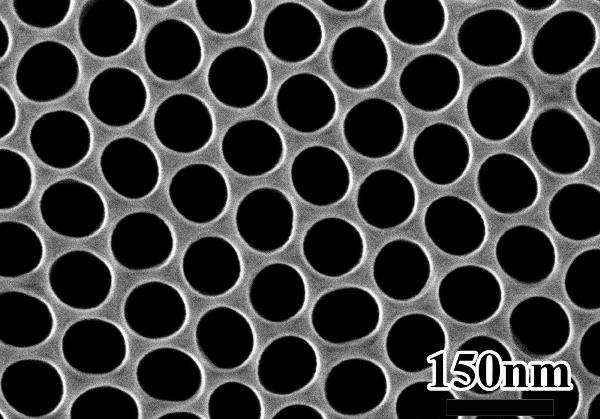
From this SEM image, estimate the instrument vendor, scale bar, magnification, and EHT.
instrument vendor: Hitachi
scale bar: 300 nm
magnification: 150 K X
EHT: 20 kV